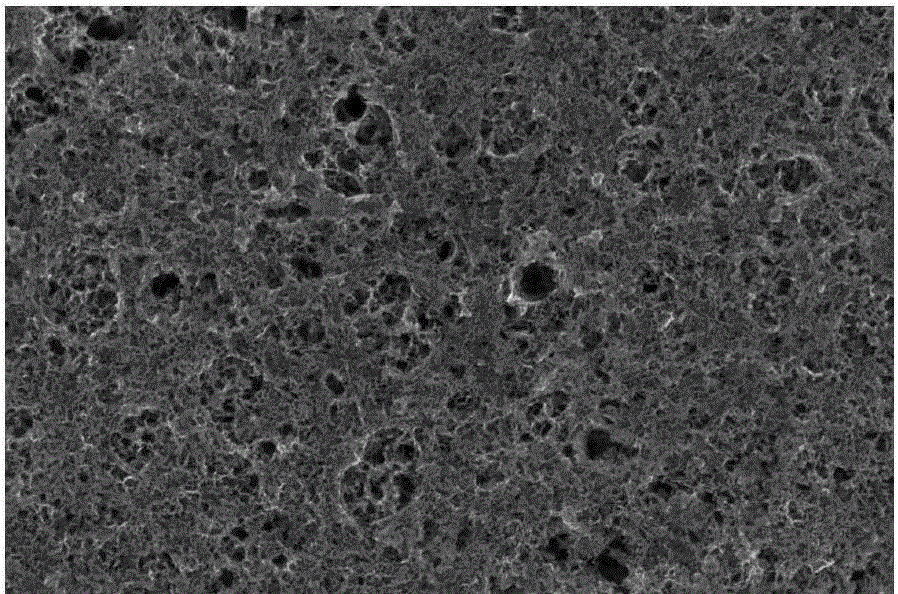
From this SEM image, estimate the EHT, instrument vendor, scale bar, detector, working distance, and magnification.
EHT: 15 kV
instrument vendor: FEI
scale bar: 500 nm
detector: TLD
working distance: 5.6 mm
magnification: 200 K X